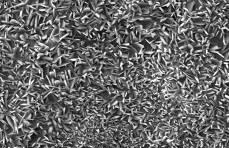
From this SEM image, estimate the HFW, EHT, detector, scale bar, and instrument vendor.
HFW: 115 µm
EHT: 20 kV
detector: SE Detector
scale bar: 50000 nm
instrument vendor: Tescan